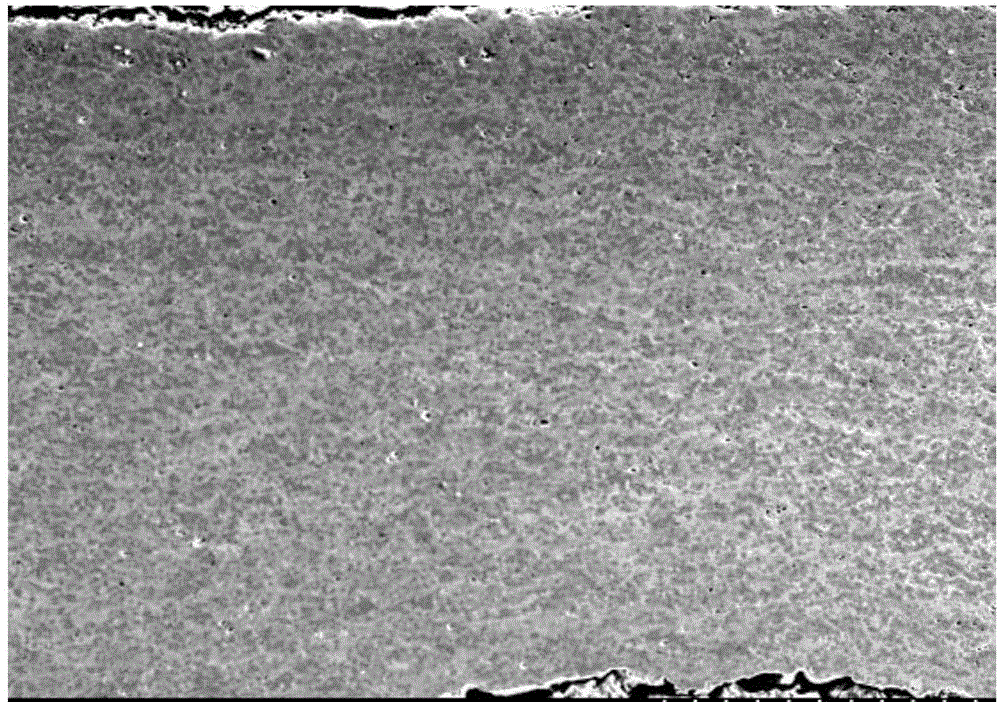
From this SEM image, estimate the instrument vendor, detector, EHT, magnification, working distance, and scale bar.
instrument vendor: Hitachi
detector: SE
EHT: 15 kV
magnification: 0.2 K X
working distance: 7 mm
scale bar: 200000 nm